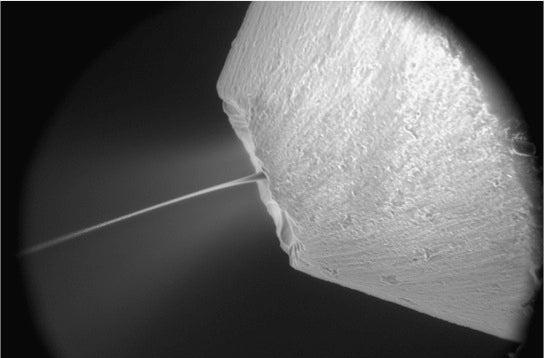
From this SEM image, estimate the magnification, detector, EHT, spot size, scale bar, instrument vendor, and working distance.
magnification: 0.15 K X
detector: GSE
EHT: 20 kV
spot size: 5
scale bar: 200000 nm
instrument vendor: FEI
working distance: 14.6 mm